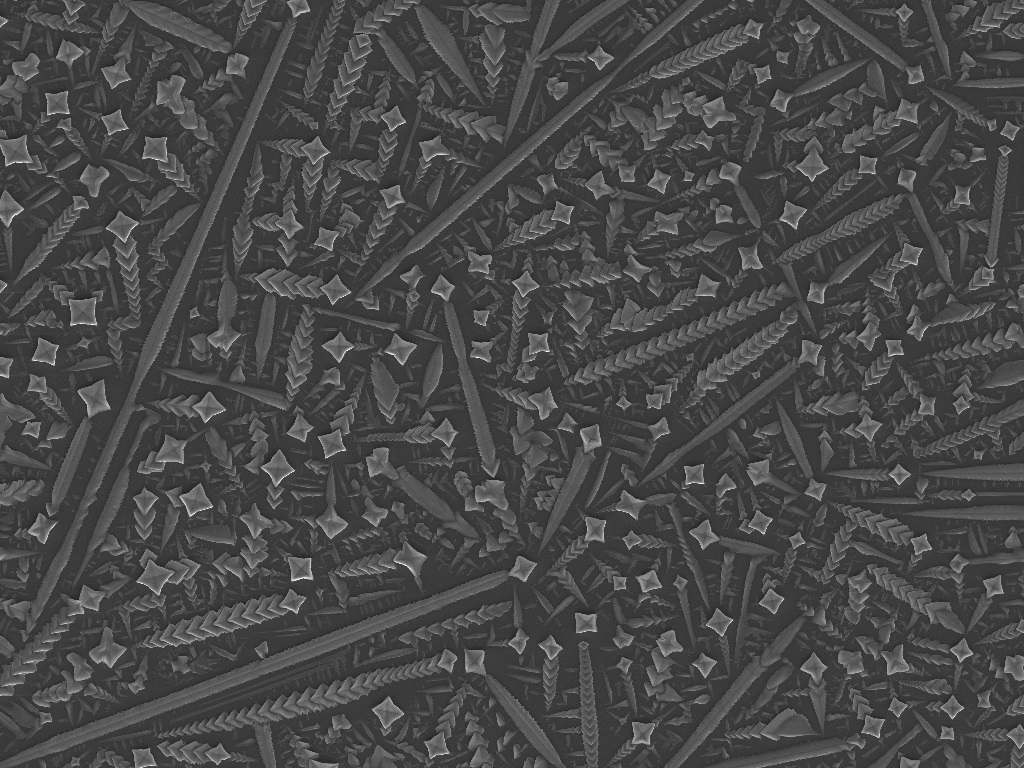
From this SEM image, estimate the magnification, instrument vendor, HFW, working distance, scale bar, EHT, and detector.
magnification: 0.588 K X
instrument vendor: Tescan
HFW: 471 µm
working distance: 15.01 mm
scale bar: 100000 nm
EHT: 20 kV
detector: SE